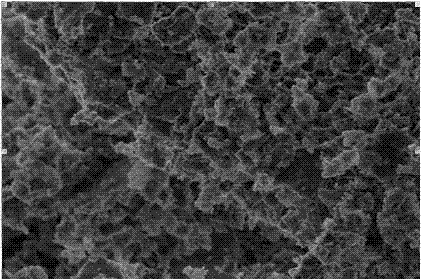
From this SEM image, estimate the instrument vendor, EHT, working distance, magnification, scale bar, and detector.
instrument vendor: Hitachi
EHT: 3 kV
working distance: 7.2 mm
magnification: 10 K X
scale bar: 5000 nm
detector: SE(M)